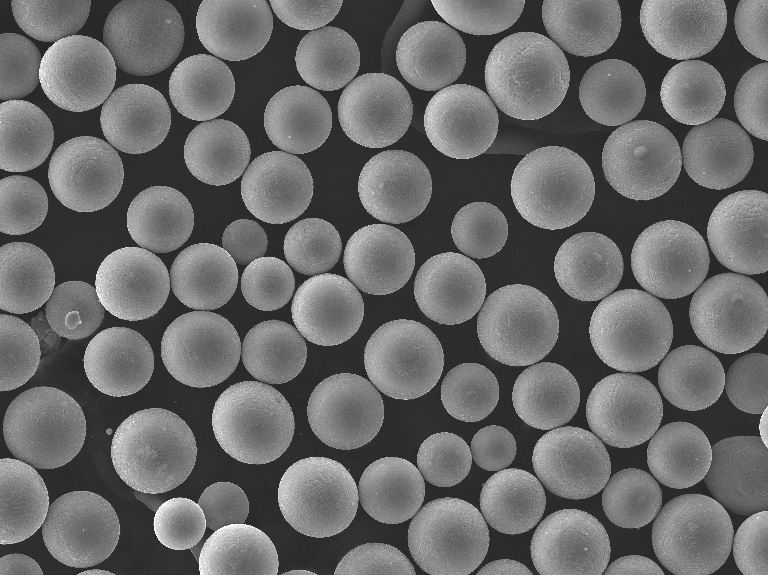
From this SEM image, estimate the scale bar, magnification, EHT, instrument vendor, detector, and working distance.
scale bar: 100000 nm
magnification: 0.5 K X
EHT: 30 kV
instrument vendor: Tescan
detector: SE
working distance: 5.46 mm